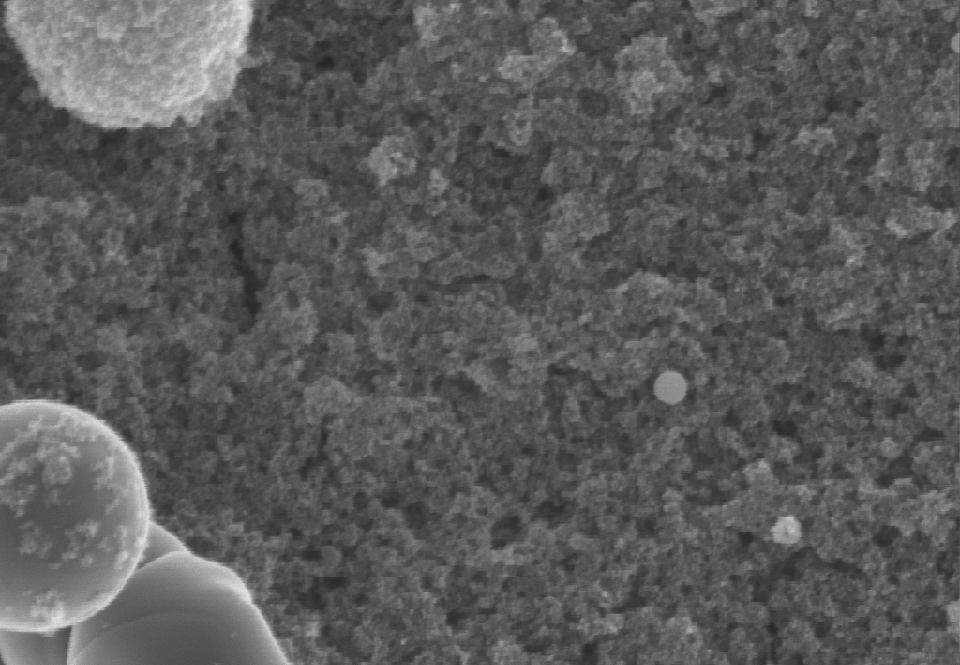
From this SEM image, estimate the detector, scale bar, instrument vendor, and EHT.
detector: InLens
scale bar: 100 nm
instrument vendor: Zeiss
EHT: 5 kV